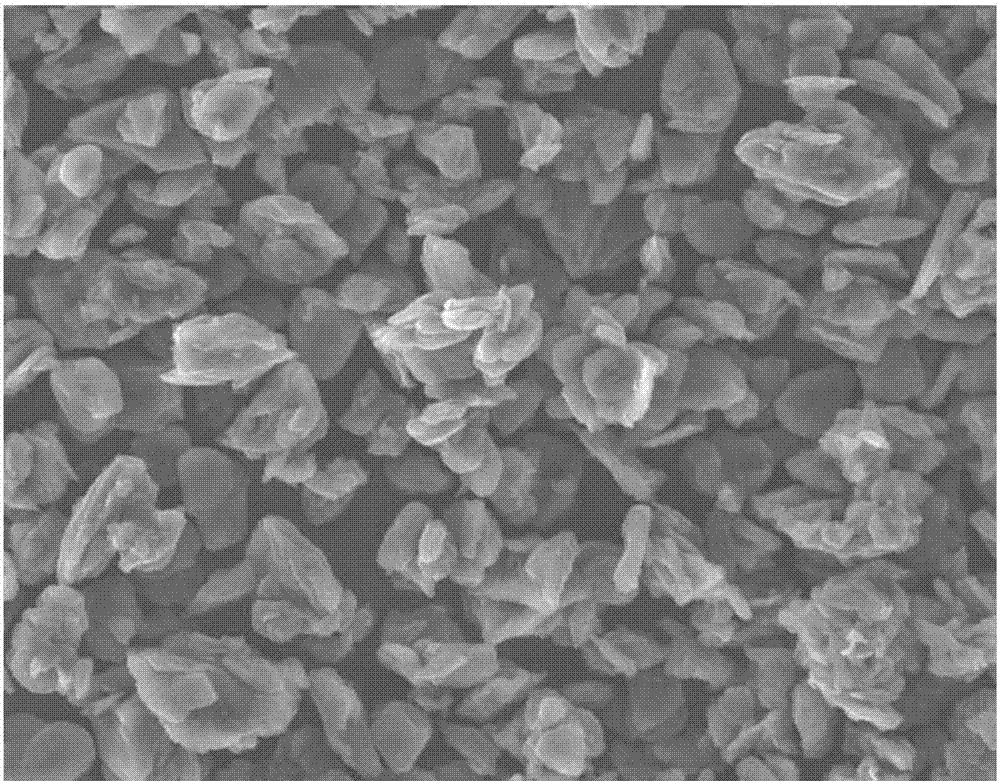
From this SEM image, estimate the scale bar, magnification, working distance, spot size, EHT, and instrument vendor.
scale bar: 50000 nm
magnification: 1 K X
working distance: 9.8 mm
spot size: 4.5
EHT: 20 kV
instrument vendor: FEI